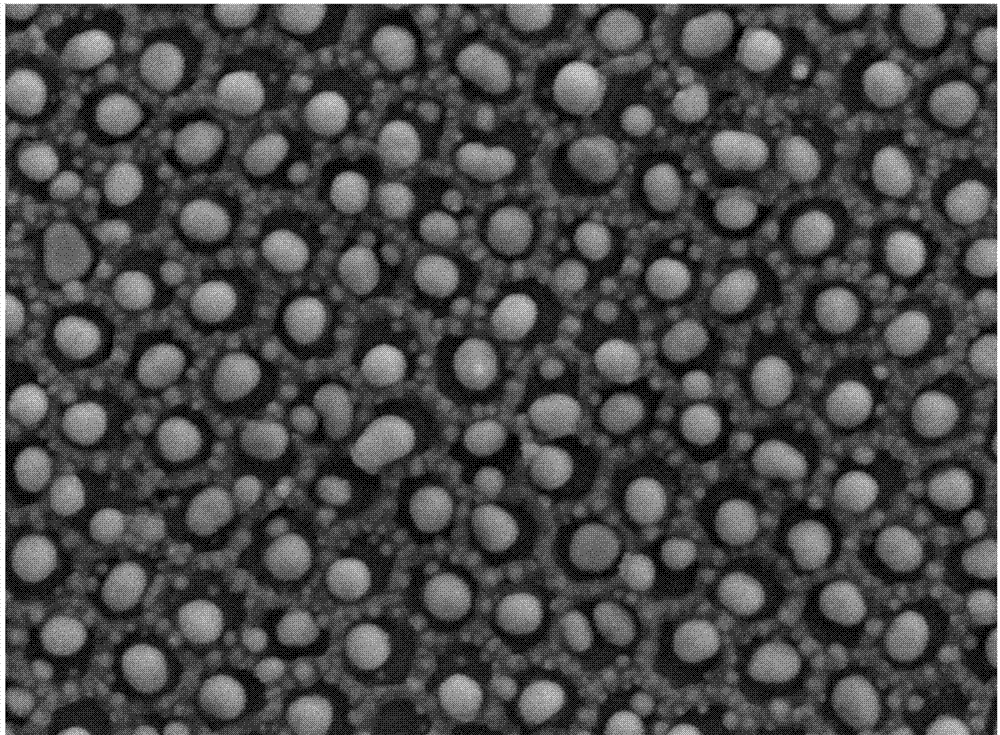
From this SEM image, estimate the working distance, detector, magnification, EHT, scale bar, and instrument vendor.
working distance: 8 mm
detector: LED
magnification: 50 K X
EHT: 5 kV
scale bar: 100 nm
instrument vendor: JEOL